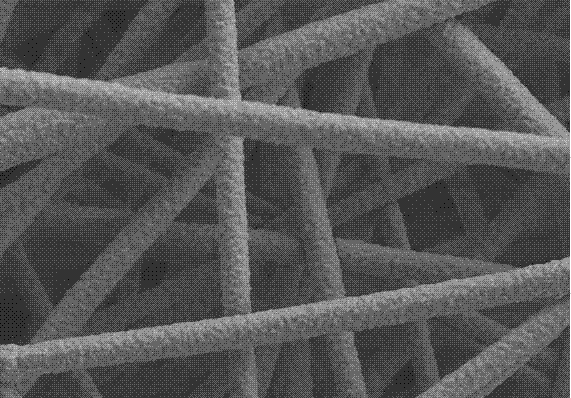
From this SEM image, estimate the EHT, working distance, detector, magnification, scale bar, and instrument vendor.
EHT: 5 kV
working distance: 18.2 mm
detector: SE(M)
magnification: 10 K X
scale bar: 5000 nm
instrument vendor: Hitachi